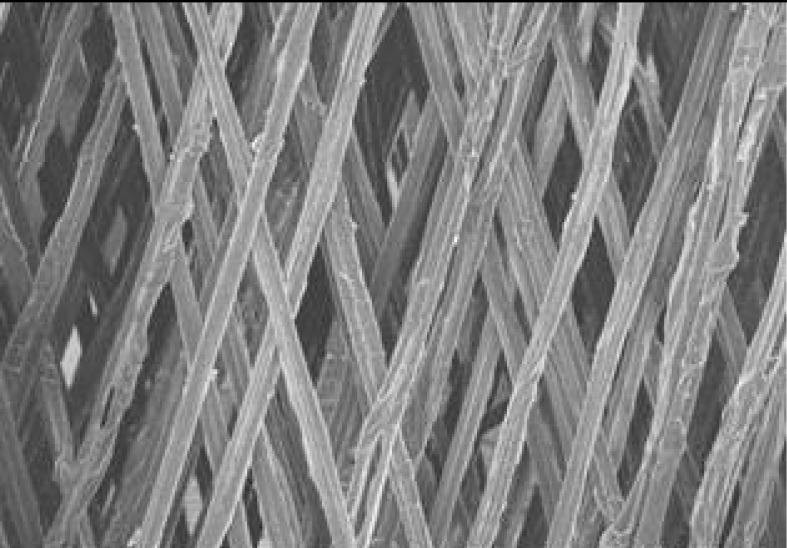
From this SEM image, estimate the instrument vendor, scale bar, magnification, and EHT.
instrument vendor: JEOL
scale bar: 500000 nm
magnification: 0.1 K X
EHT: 15 kV